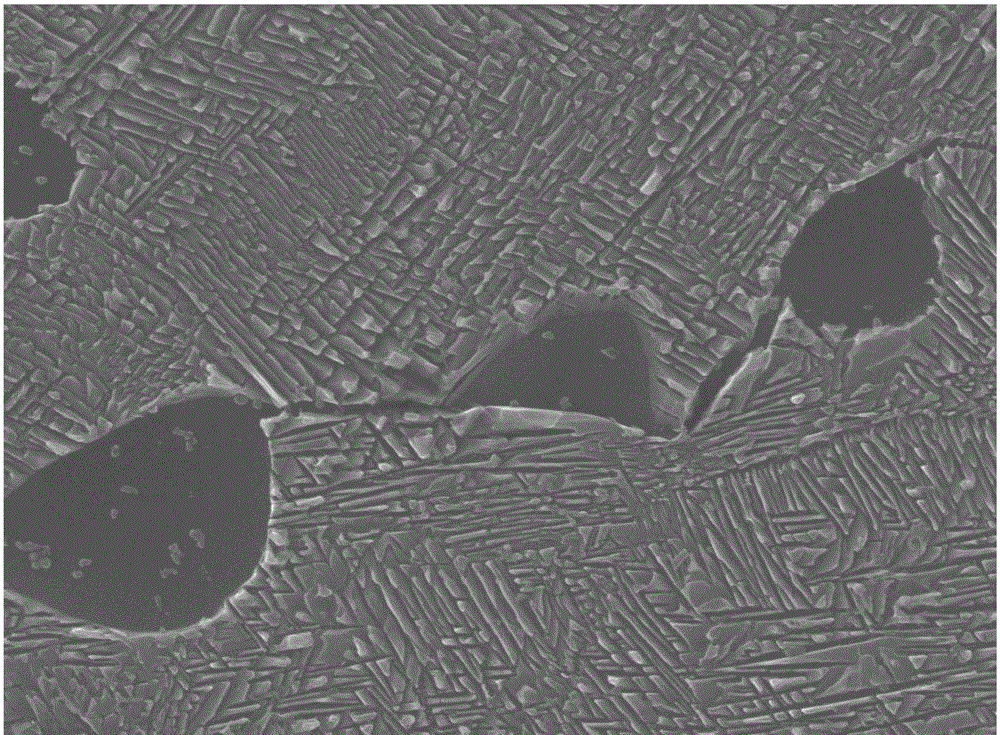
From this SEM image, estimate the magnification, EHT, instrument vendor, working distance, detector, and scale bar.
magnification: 20 K X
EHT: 15 kV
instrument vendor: JEOL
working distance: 7.9 mm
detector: SEI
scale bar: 1000 nm